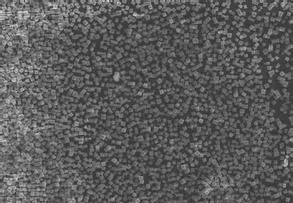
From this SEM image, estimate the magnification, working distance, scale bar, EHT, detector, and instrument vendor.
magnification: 0.4 K X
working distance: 11.6 mm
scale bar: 100000 nm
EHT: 15 kV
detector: SE(M)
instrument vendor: Hitachi